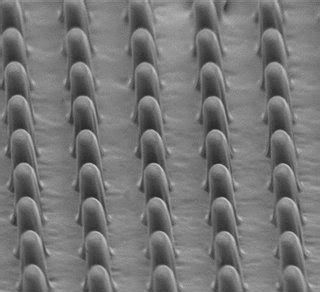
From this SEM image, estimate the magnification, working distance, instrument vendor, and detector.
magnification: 5 K X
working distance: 12.6 mm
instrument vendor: Hitachi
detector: SE(M)